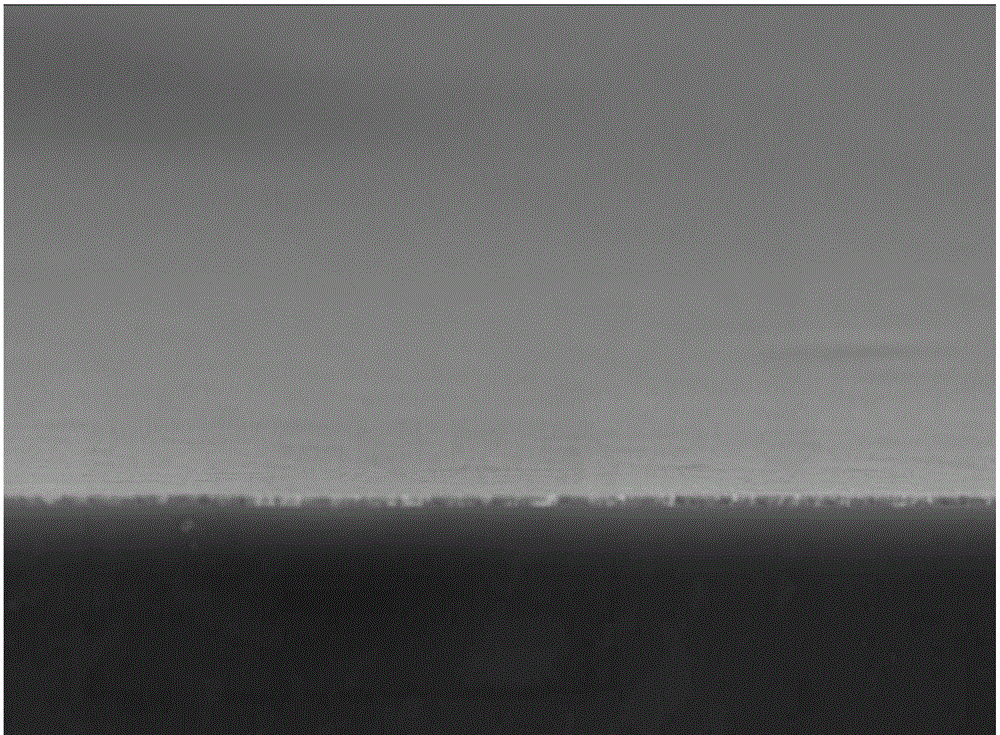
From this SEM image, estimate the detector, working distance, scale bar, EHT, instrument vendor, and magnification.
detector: LED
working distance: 9.7 mm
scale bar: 1000 nm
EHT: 10 kV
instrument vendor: JEOL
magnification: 10 K X